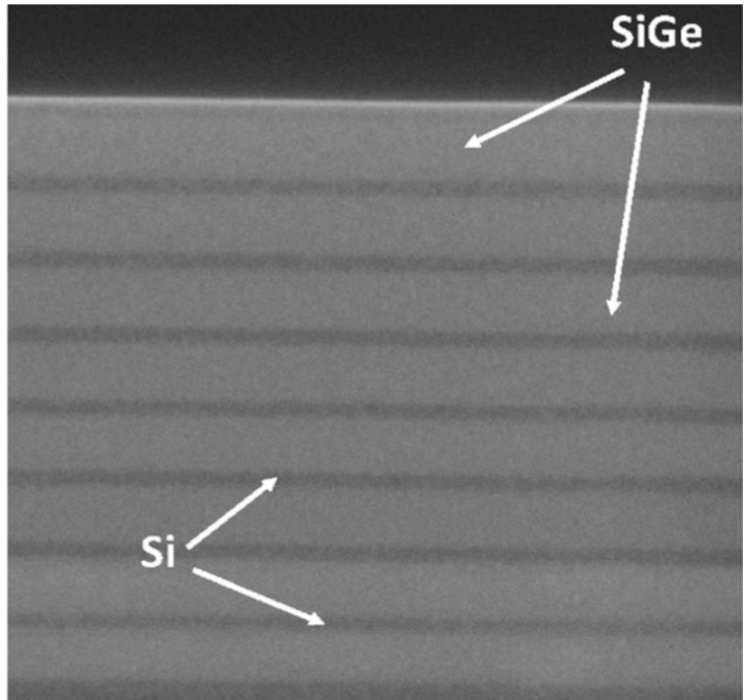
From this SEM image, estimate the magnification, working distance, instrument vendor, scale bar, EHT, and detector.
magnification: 150 K X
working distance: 0.3 mm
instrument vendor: Hitachi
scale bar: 300 nm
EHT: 3 kV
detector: SE